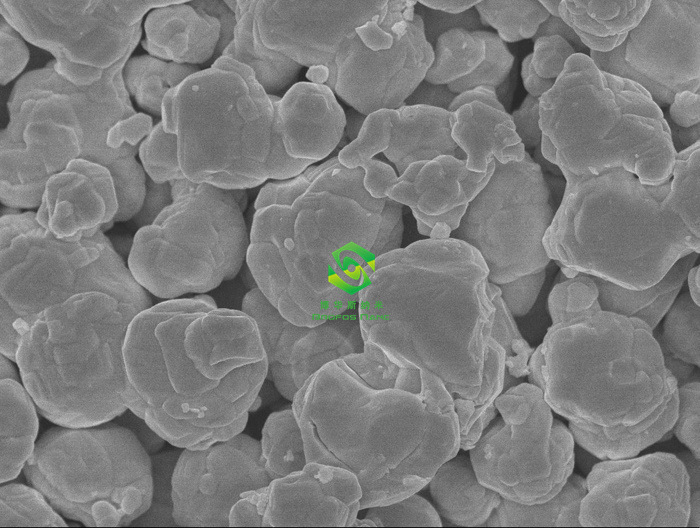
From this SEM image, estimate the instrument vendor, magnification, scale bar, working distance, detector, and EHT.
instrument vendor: JEOL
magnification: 10 K X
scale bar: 1000 nm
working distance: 8 mm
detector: SEI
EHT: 10 kV